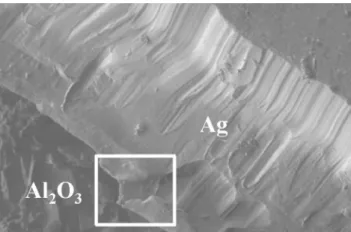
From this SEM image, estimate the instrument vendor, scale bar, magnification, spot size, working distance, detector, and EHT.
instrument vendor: FEI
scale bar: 200000 nm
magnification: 0.181 K X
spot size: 5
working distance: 19.1 mm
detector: GSE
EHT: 20 kV